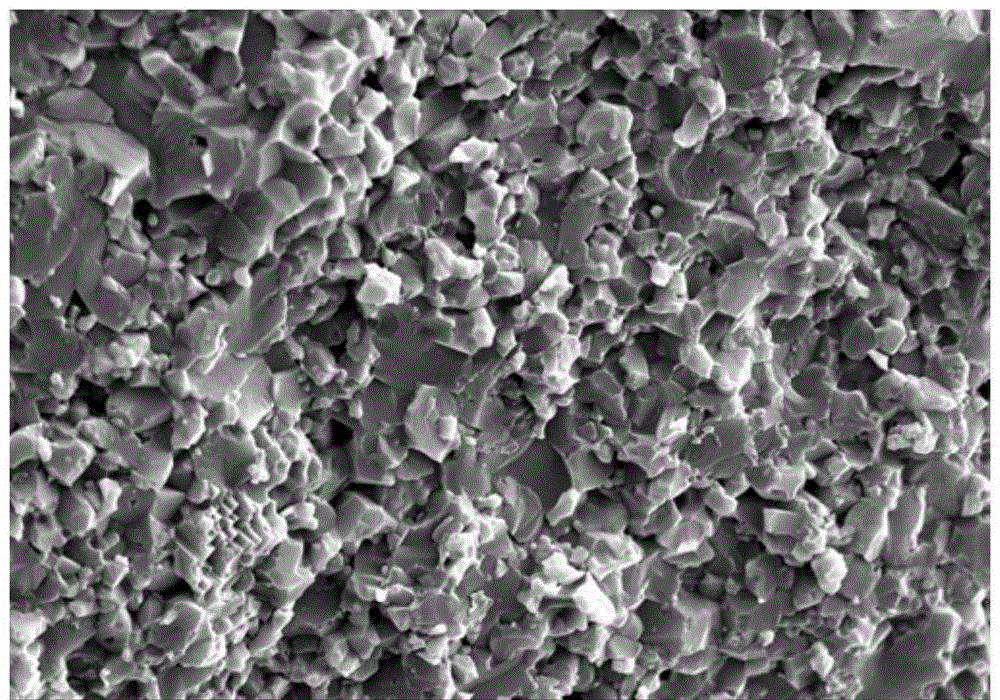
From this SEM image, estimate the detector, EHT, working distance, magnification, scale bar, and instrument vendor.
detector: SE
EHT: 15 kV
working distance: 6 mm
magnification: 4 K X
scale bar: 10000 nm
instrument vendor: Hitachi